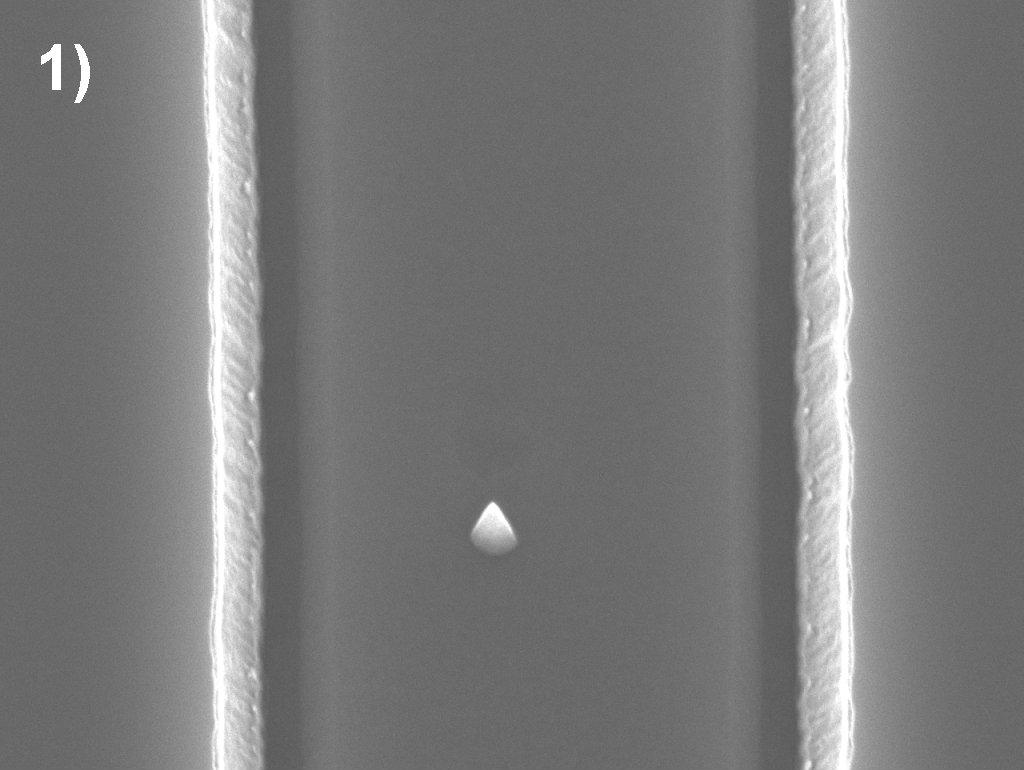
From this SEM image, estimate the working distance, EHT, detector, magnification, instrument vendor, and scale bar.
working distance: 10 mm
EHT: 15 kV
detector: SEI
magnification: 20 K X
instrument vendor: JEOL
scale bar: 1000 nm